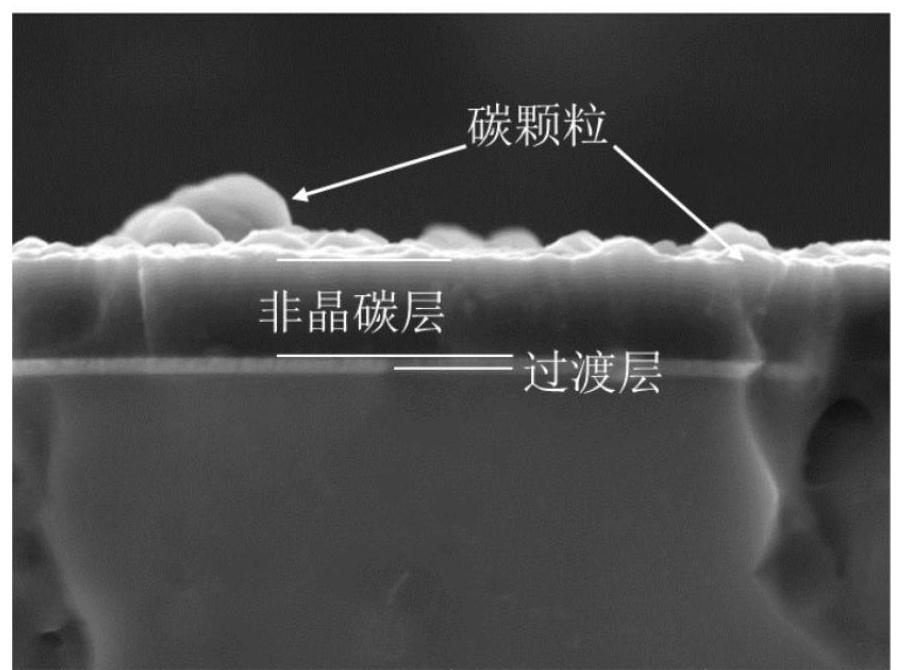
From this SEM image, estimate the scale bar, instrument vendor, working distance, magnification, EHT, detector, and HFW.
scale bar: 2000 nm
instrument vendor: Tescan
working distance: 12.03 mm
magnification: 40 K X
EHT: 7 kV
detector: SE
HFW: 6.92 µm